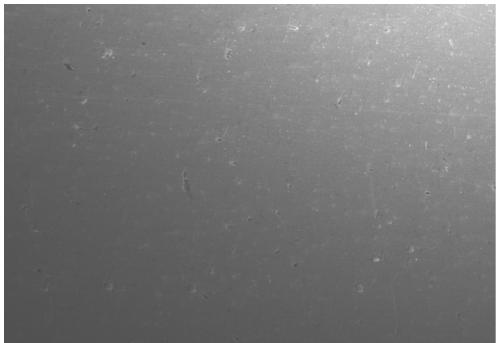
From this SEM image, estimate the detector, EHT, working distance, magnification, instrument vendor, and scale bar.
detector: SE(U)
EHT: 5 kV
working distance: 8.2 mm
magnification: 0.5 K X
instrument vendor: Hitachi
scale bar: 100000 nm